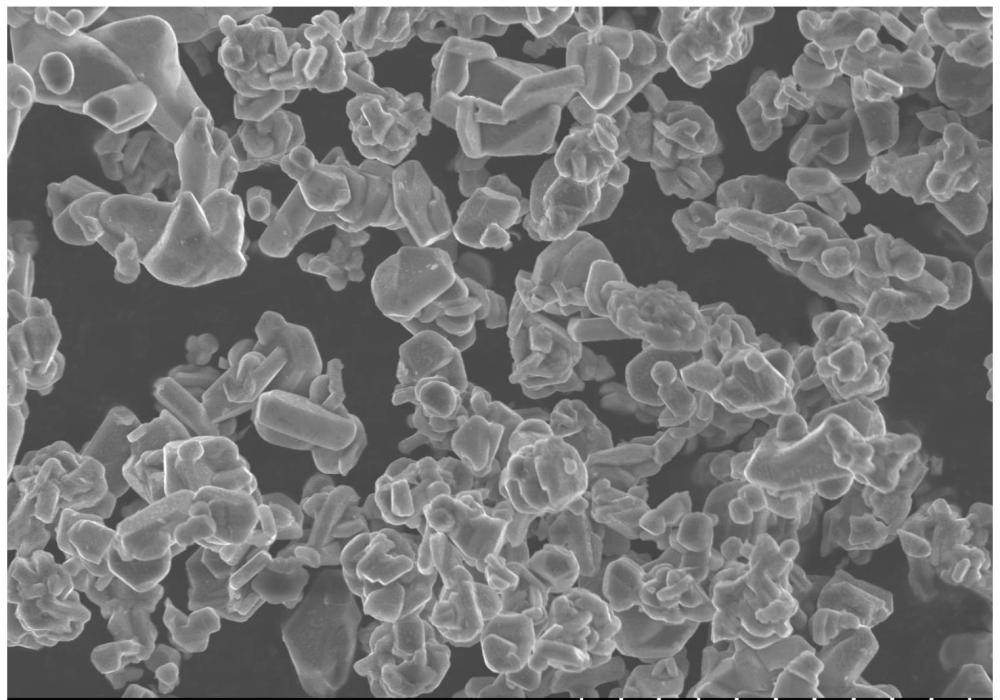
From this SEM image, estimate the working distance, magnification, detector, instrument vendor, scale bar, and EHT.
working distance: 8.3 mm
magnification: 2.5 K X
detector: SE(UL)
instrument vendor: Hitachi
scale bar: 20000 nm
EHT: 10 kV